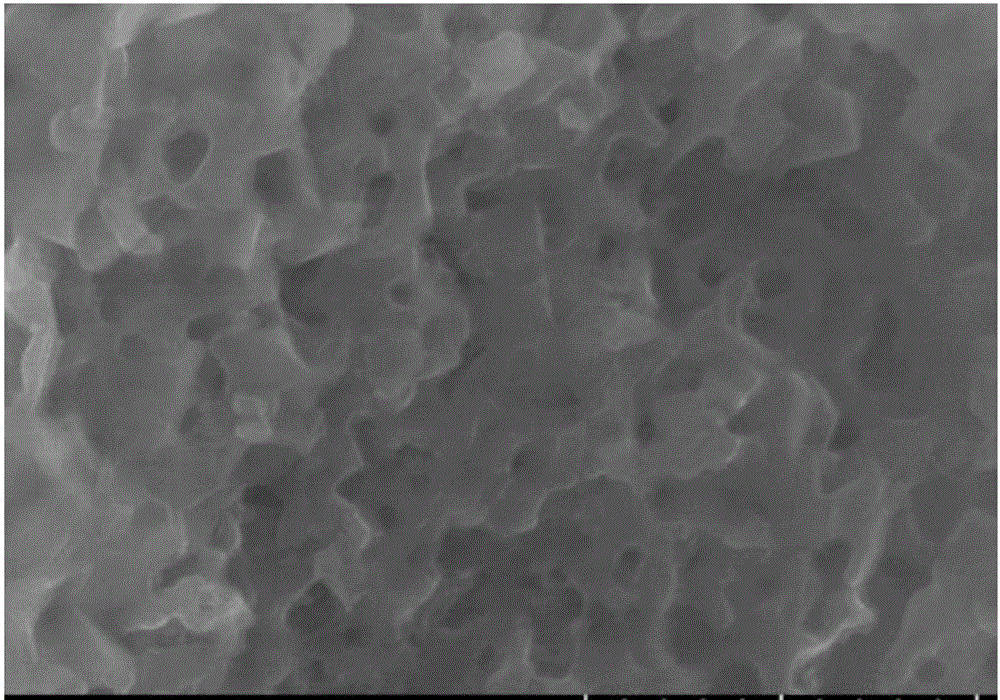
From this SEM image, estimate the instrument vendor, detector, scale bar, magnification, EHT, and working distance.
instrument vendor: Hitachi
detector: SE(UL)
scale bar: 1000 nm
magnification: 50 K X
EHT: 15 kV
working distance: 9.4 mm